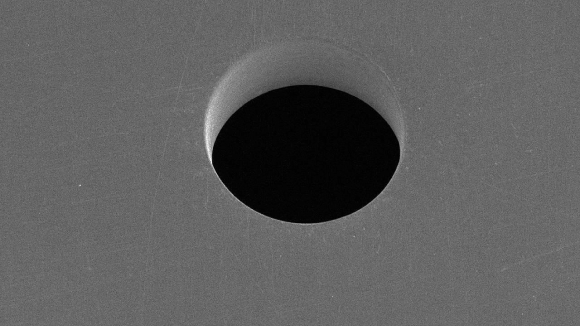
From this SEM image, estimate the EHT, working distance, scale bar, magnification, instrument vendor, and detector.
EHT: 5 kV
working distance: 21.8 mm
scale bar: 50000 nm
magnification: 1 K X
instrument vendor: Hitachi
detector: SE